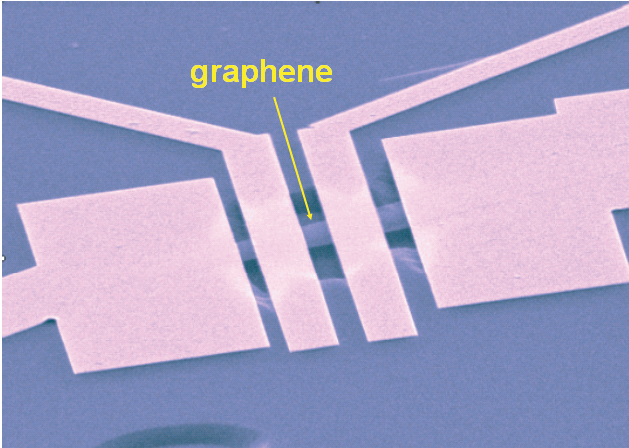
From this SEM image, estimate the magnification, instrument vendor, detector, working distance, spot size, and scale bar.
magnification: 6.22 K X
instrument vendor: FEI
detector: SE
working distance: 10.6 mm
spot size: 1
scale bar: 2000 nm